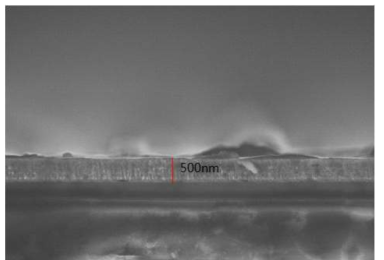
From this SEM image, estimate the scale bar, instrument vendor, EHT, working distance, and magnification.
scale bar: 3000 nm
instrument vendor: Hitachi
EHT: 5 kV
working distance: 10.4 mm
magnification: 15 K X